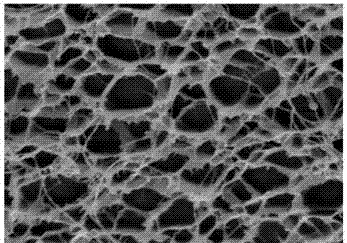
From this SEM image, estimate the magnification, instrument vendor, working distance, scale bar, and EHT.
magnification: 5 K X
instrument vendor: FEI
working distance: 8.1 mm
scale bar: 10000 nm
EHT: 15 kV